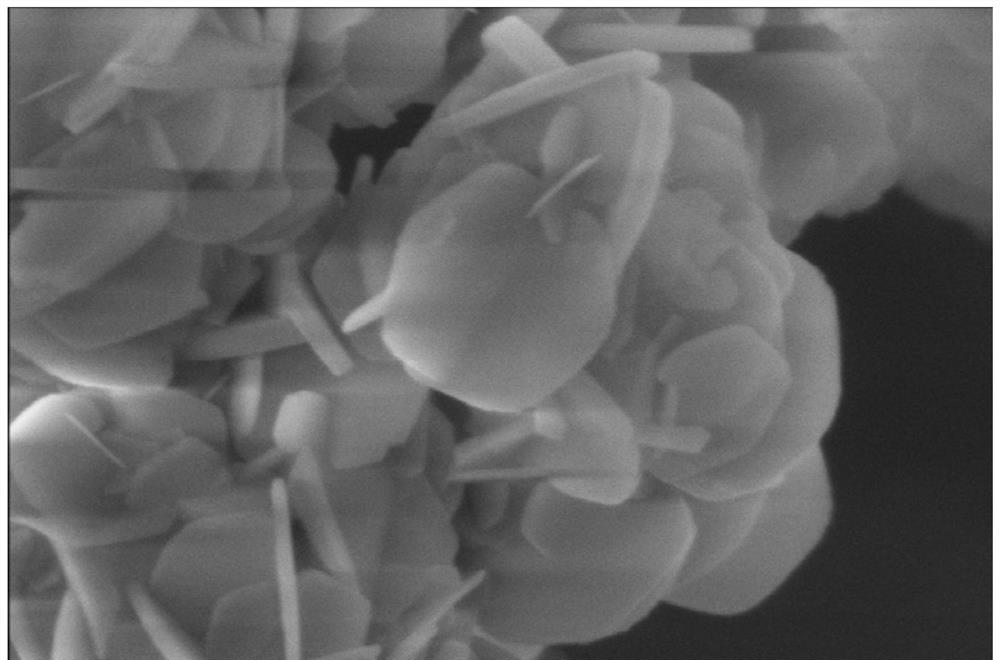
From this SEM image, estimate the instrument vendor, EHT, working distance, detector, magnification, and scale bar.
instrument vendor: FEI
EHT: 5 kV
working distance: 4 mm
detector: TLD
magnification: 200 K X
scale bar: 500 nm